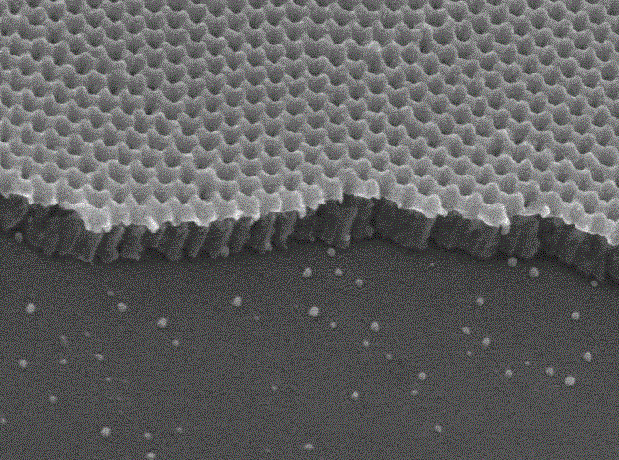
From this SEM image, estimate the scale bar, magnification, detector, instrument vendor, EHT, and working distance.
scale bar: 100 nm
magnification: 50 K X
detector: SEI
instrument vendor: JEOL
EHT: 15 kV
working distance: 14.9 mm